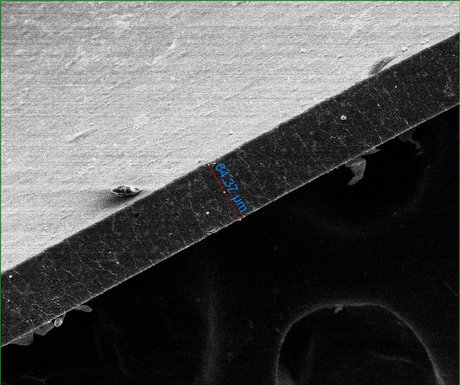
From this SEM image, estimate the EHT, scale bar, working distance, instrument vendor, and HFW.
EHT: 15 kV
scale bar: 200000 nm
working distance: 11.3 mm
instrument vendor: FEI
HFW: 469 µm